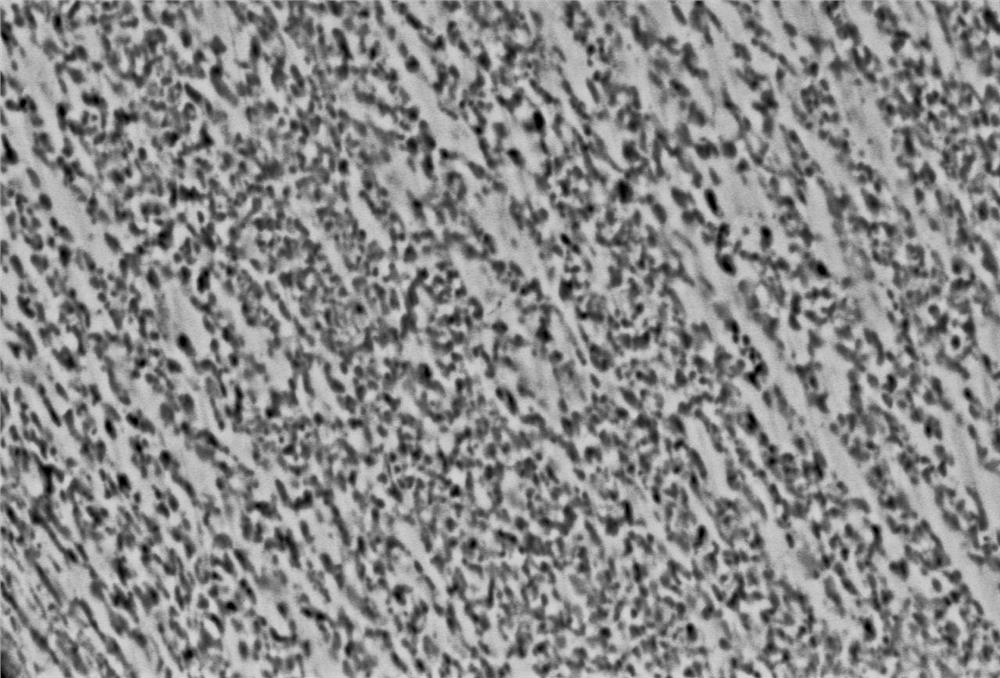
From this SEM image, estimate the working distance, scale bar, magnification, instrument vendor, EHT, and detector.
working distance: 13 mm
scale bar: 5000 nm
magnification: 3 K X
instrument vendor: JEOL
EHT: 20 kV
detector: BEC